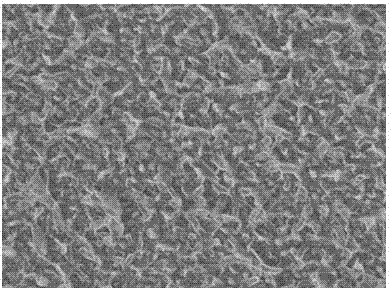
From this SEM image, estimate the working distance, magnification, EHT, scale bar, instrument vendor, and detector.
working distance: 8.3 mm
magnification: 20 K X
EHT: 5 kV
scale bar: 1000 nm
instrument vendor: JEOL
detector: LEI